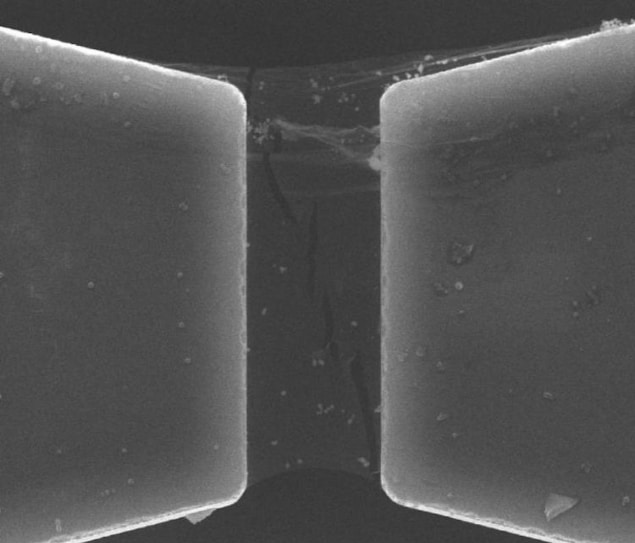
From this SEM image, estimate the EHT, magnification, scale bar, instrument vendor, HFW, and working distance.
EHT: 20 kV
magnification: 10 K X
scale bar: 10000 nm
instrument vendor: FEI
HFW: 29.8 µm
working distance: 8.2 mm